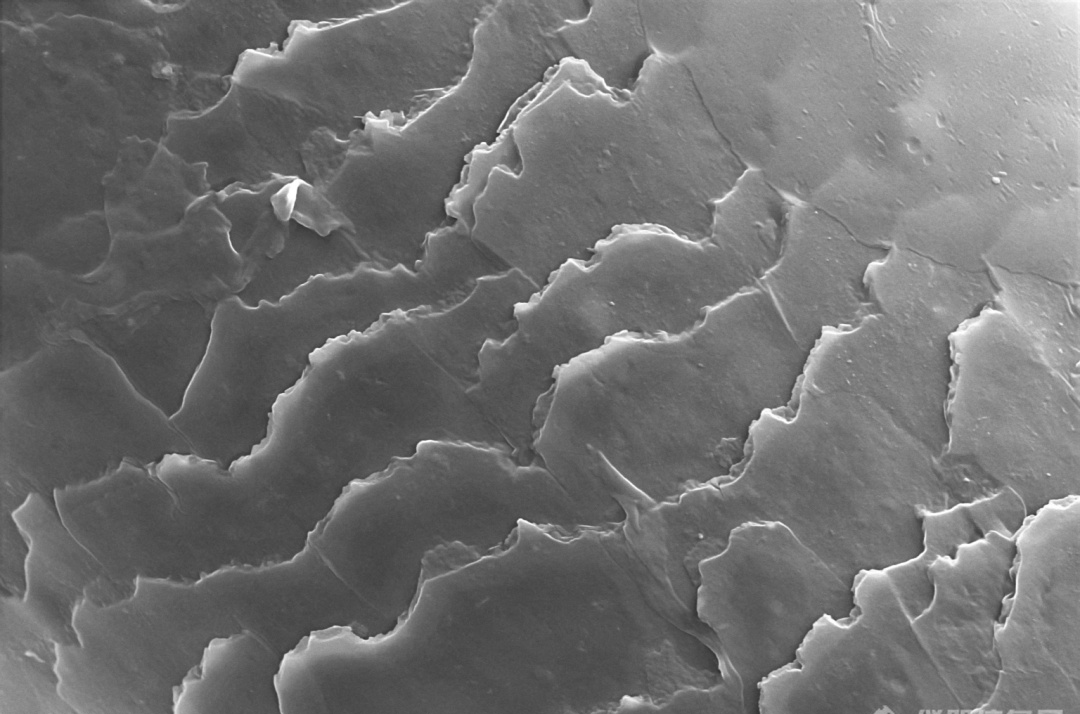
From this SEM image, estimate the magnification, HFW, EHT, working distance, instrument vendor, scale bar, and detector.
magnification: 2 K X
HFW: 63.5 µm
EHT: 15 kV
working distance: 8.1 mm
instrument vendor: Thermo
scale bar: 10000 nm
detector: ETD